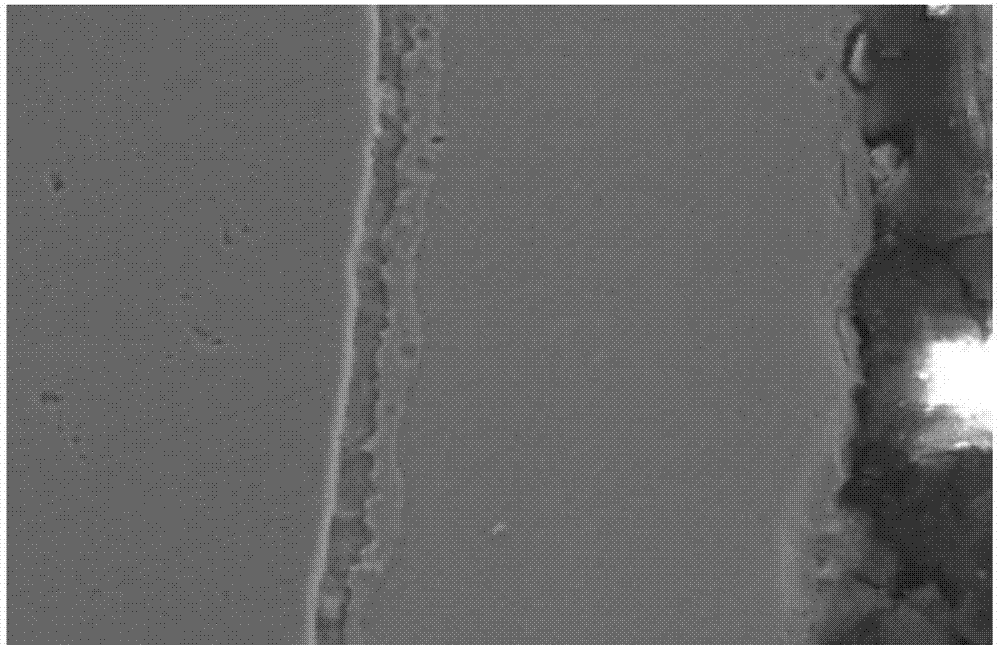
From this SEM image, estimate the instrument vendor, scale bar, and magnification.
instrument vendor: JEOL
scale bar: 10000 nm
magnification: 1 K X